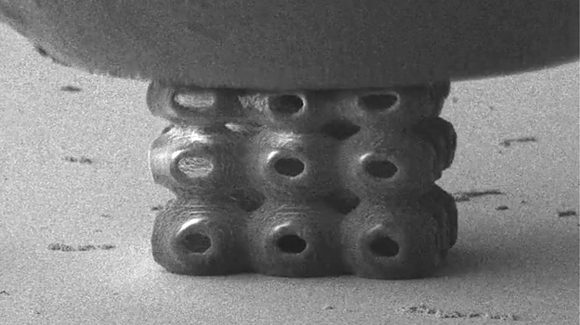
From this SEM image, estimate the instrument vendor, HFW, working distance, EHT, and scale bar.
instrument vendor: FEI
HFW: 59.2 µm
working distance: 7 mm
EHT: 2 kV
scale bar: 20000 nm